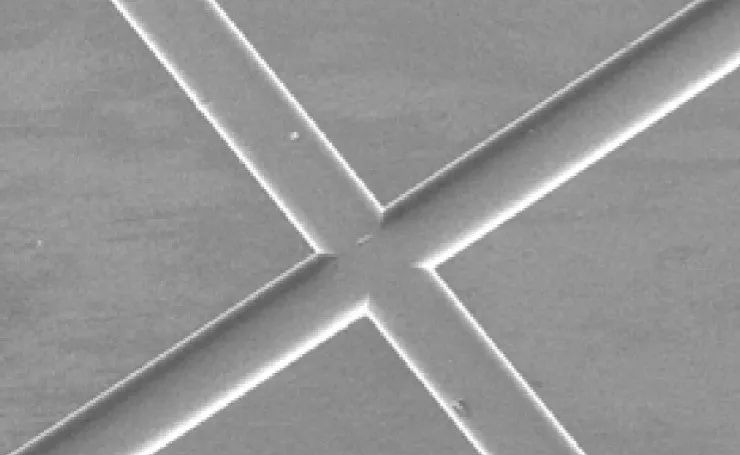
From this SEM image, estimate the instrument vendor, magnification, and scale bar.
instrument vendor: FEI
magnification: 0.1 K X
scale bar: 200000 nm